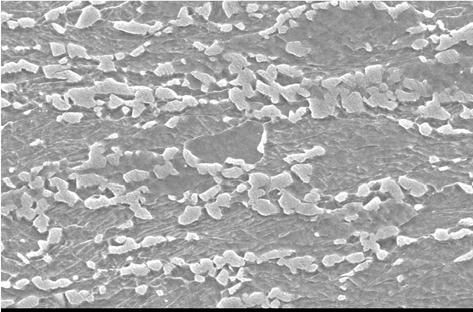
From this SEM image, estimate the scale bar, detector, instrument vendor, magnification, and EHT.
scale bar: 5000 nm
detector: SEI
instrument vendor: JEOL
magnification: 5 K X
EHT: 15 kV